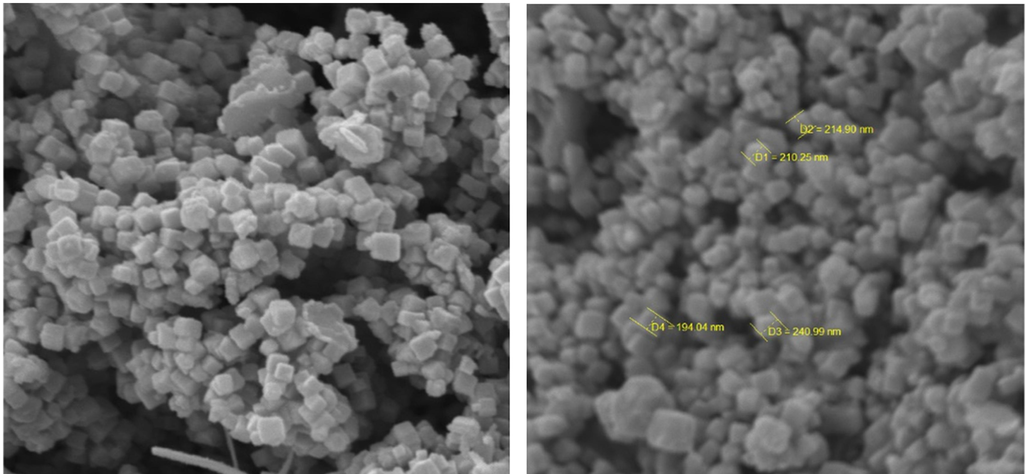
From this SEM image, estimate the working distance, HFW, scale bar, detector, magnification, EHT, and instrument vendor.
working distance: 5.02 mm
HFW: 5.19 µm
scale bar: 1000 nm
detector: SE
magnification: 40 K X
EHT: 10 kV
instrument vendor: Tescan